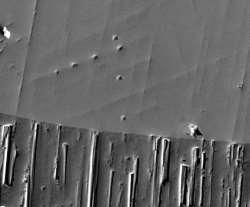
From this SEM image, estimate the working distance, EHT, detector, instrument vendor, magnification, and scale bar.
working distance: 10 mm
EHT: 20 kV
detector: ETD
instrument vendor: FEI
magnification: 1.2 K X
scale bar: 50000 nm